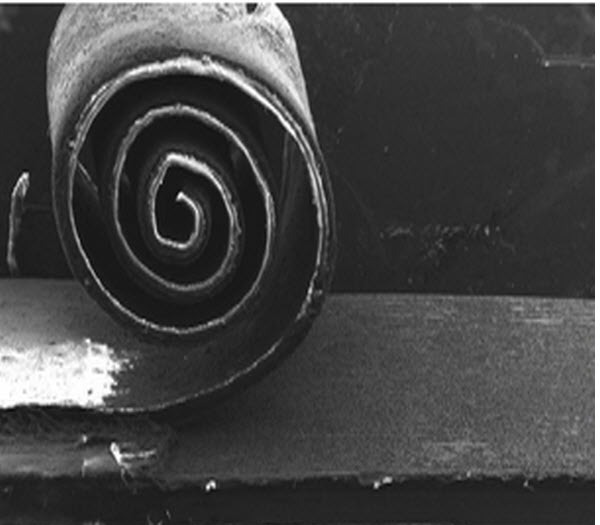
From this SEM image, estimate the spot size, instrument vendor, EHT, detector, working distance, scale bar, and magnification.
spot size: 3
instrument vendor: FEI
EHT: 15 kV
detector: SE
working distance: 13.8 mm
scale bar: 1000000 nm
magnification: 0.03 K X